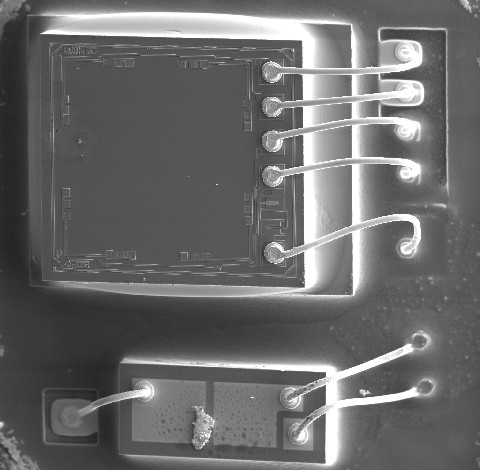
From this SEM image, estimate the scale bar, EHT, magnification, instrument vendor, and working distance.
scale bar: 200000 nm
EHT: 20 kV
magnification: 0.05 K X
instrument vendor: FEI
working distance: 17 mm